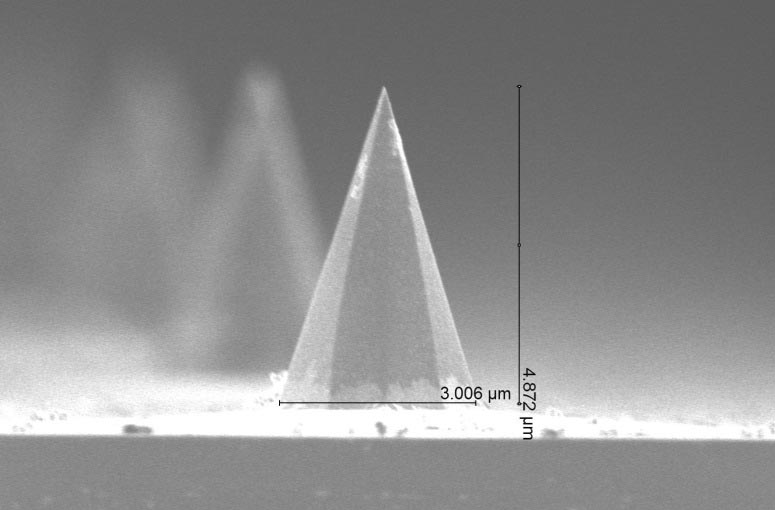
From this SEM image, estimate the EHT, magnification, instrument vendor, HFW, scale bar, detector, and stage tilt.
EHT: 2 kV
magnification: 35 K X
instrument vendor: FEI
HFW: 11.8 µm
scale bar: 3000 nm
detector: TLD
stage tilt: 43°